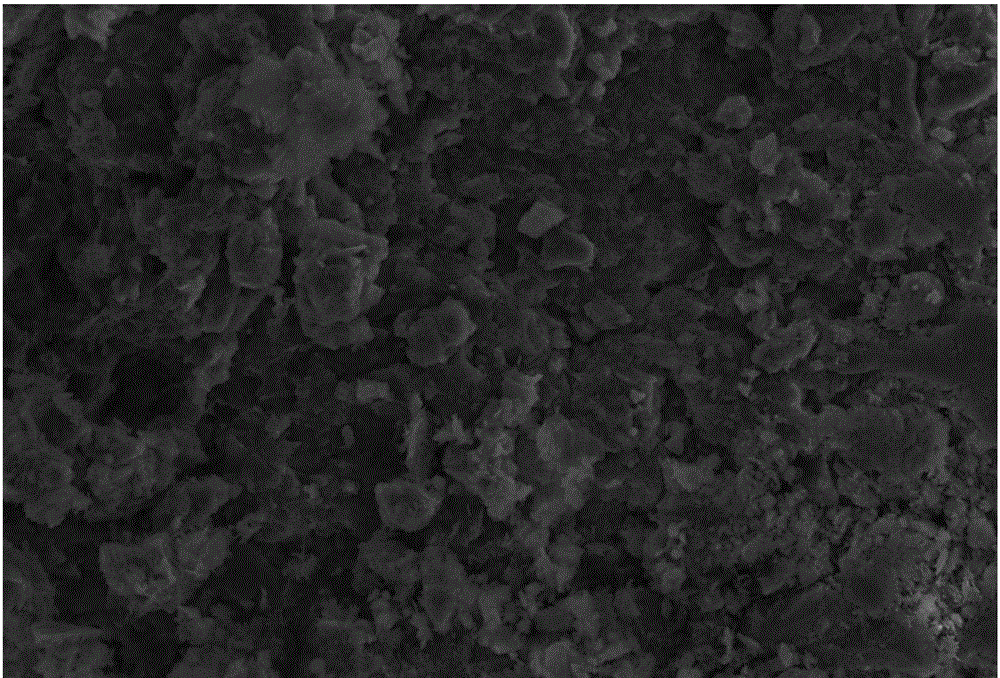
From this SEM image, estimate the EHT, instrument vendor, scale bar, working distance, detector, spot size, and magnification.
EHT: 15 kV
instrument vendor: JEOL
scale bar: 10000 nm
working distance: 11 mm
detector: SEI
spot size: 35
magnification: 1 K X